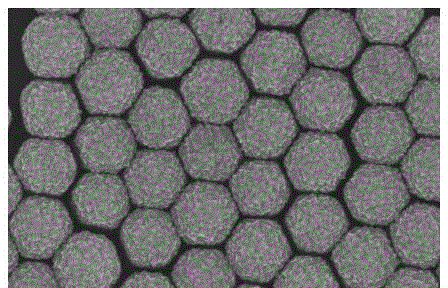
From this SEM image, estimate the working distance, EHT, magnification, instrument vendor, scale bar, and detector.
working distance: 11 mm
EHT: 15 kV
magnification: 40 K X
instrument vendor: Hitachi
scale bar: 1000 nm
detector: SE(M)